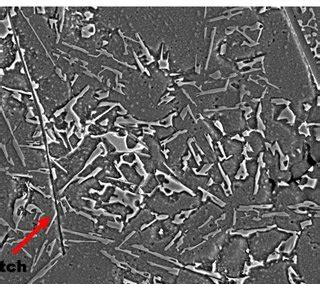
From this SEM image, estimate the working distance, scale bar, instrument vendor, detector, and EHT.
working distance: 5.4 mm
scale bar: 10000 nm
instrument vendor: Zeiss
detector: SE2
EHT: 10 kV